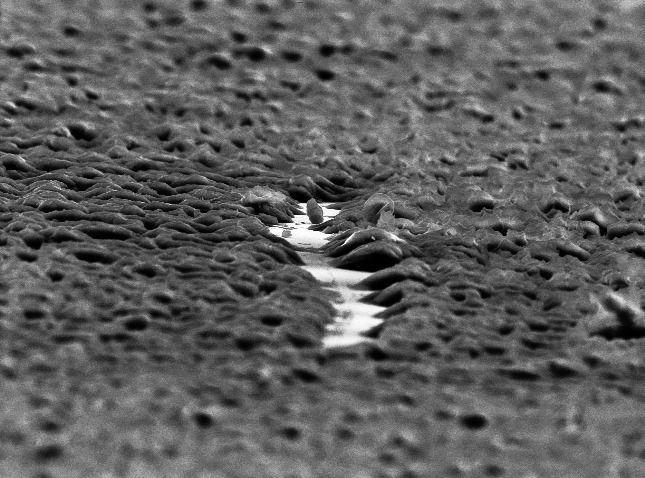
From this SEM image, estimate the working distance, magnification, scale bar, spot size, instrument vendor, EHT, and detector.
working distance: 18.6 mm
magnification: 2.096 K X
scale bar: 10000 nm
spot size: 3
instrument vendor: FEI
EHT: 10 kV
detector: SE